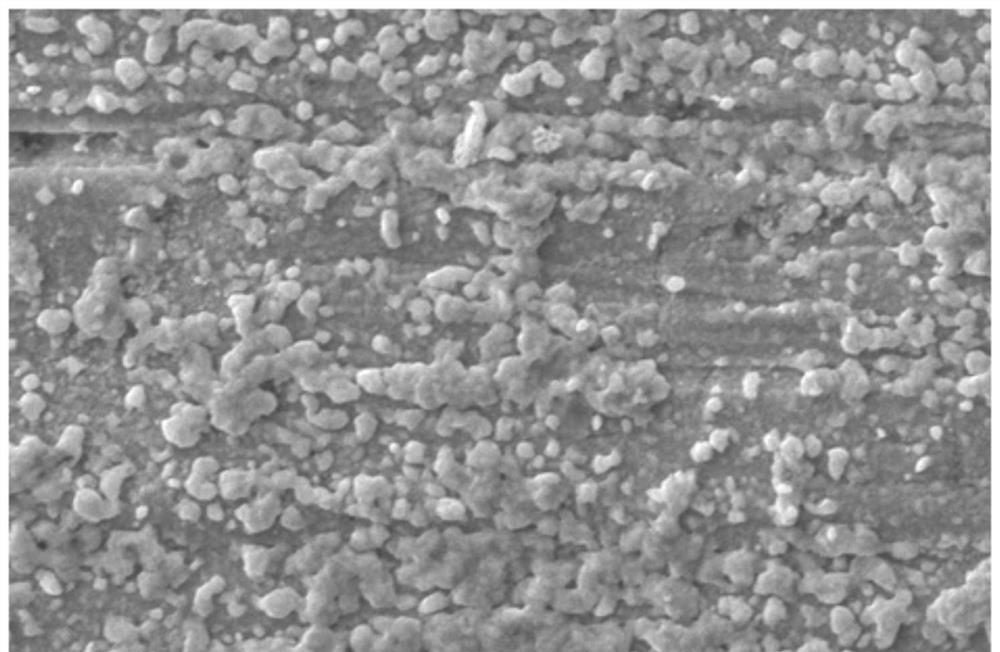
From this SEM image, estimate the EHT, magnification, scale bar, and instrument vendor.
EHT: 20 kV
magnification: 3 K X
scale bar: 5000 nm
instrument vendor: JEOL